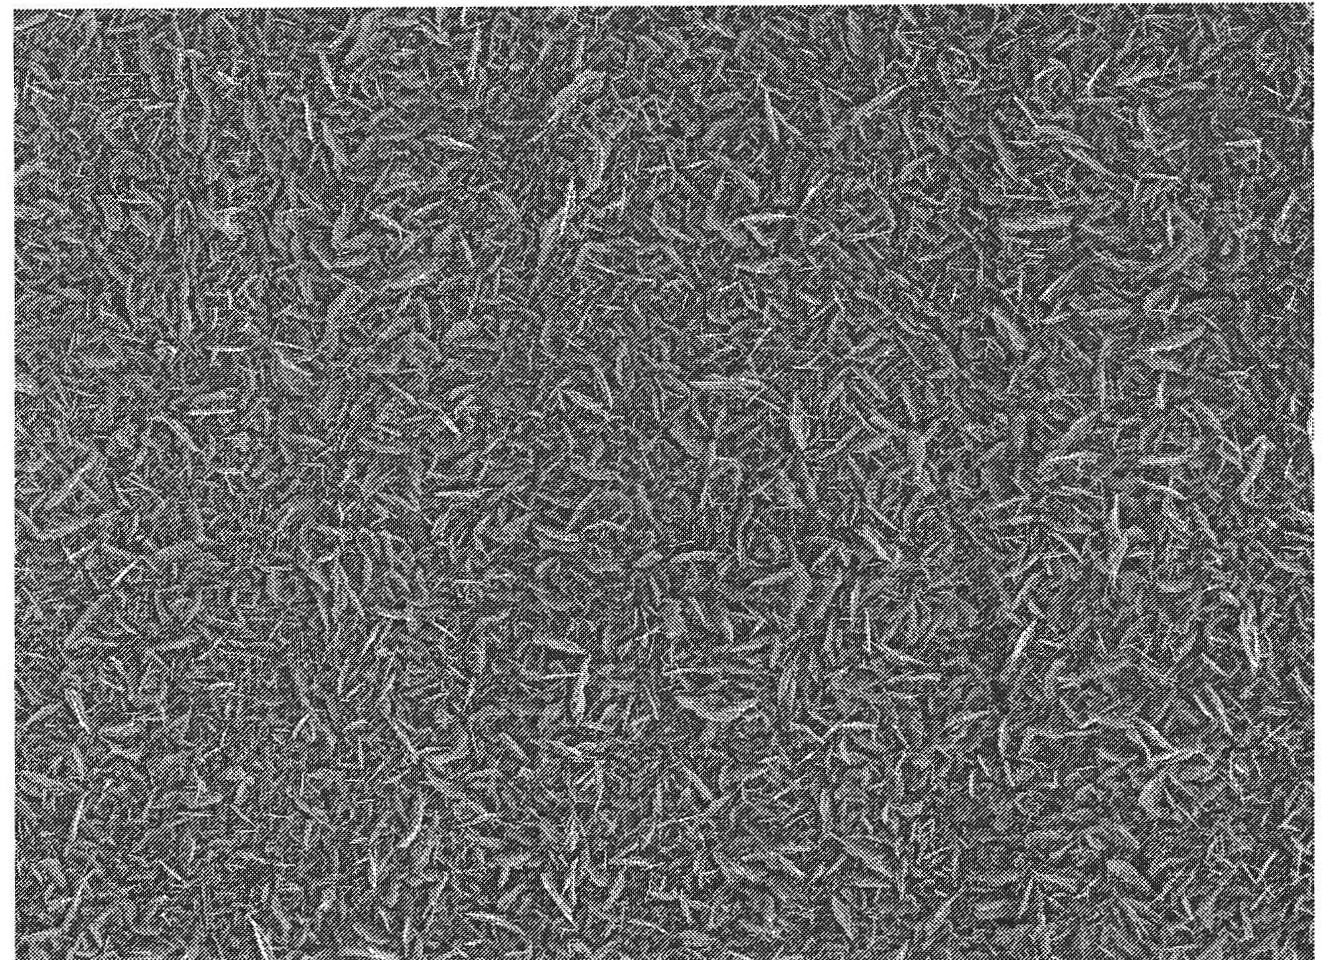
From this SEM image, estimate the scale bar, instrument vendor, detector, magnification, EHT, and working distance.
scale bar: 1000 nm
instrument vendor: JEOL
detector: SEI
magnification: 10 K X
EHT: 5 kV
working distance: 8.1 mm